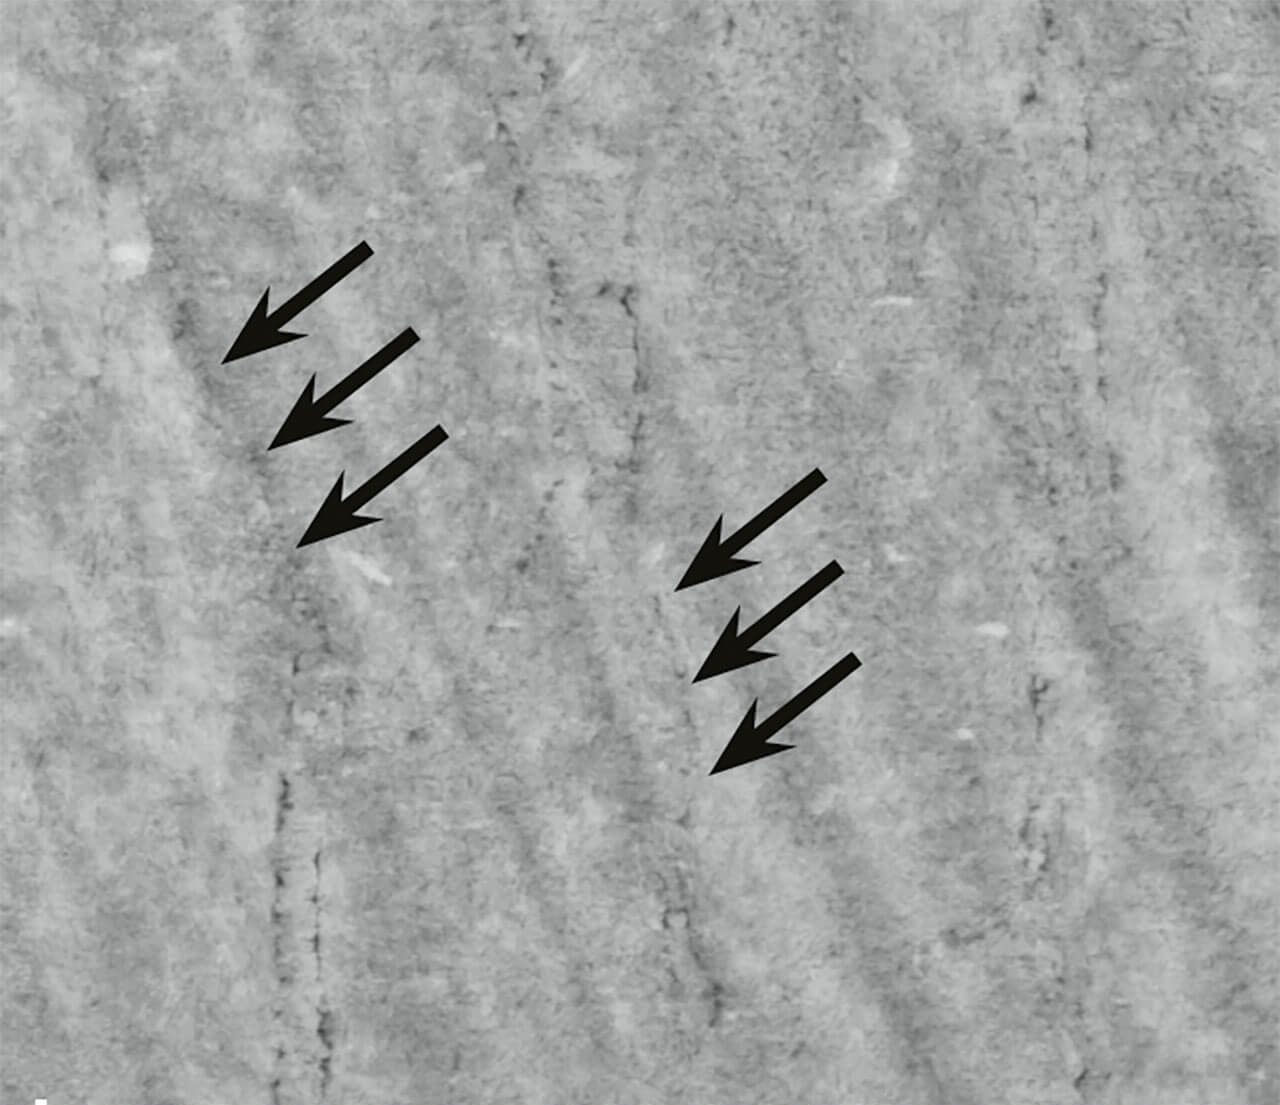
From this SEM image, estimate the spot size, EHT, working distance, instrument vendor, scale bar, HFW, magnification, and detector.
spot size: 4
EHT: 10 kV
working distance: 10 mm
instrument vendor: FEI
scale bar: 2000 nm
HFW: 10.82 µm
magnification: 25 K X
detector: SE